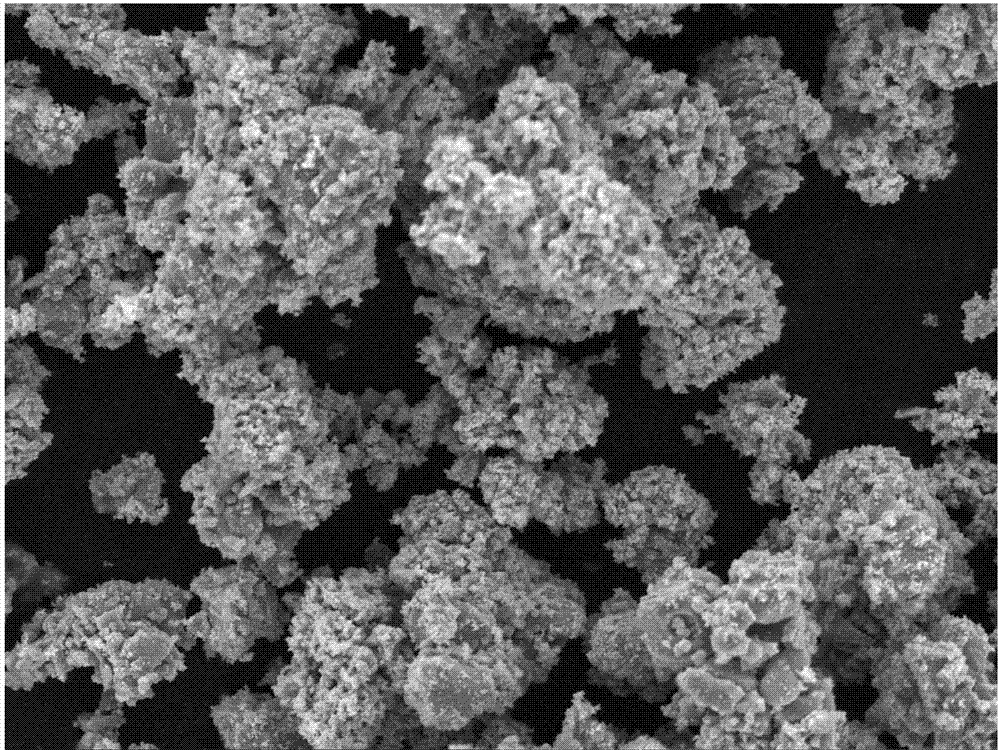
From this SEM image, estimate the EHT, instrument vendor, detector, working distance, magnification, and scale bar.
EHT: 20 kV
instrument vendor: Tescan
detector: SE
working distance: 10.42 mm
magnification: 2 K X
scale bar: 20000 nm